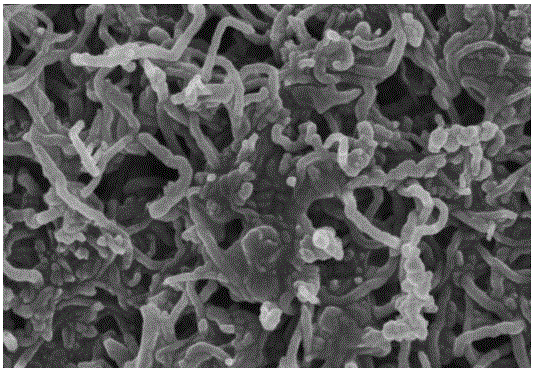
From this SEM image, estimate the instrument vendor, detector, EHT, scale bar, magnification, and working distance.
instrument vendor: Hitachi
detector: SE(M)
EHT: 3 kV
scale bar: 500 nm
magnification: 60 K X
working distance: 9.1 mm